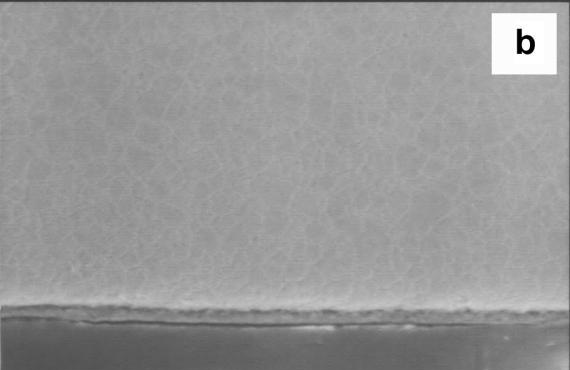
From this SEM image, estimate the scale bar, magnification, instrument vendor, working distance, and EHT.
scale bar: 10000 nm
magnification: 0.5 K X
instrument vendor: JEOL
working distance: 39 mm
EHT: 20 kV